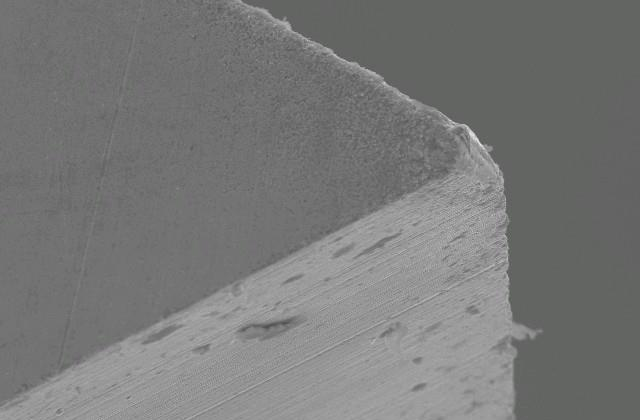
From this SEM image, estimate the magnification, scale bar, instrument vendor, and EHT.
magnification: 0.5 K X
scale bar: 50000 nm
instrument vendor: JEOL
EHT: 20 kV